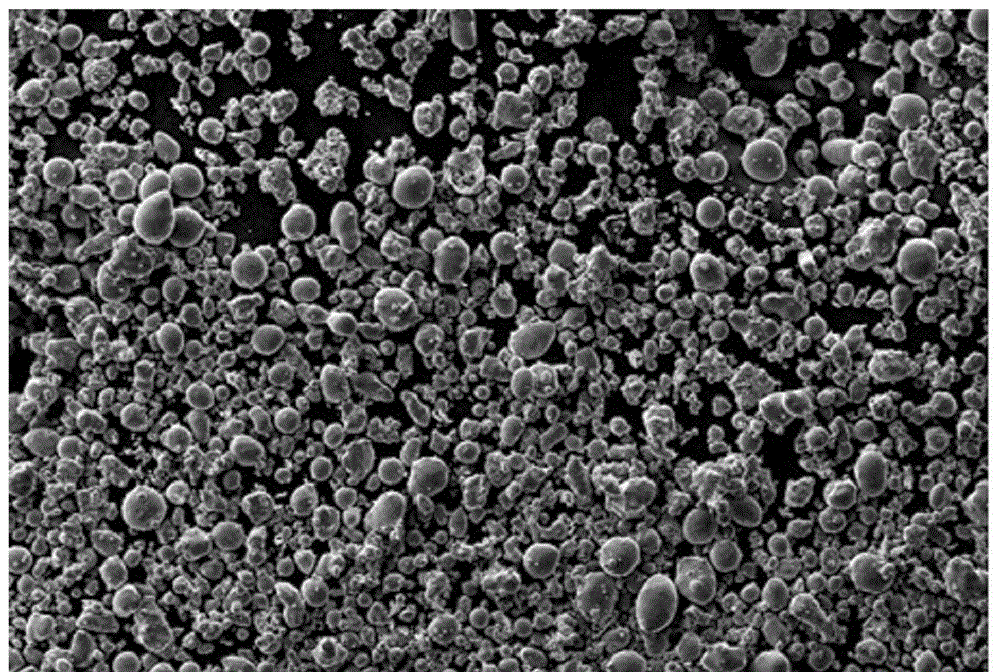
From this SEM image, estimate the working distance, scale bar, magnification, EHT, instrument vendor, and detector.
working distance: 8 mm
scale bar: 20000 nm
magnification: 0.2 K X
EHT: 20 kV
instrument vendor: Zeiss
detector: SE1